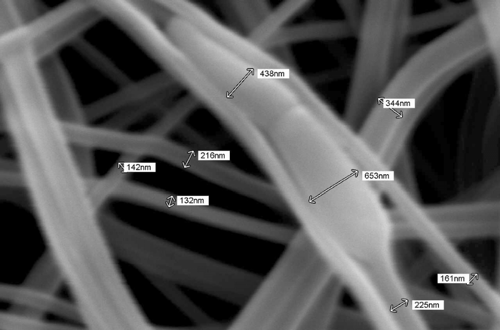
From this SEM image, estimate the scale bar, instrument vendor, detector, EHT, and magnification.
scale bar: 1000 nm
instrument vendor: JEOL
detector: SEI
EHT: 15 kV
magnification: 22 K X